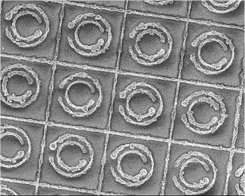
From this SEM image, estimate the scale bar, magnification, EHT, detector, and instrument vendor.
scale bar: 10000 nm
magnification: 0.95 K X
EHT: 5 kV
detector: SEI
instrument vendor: JEOL